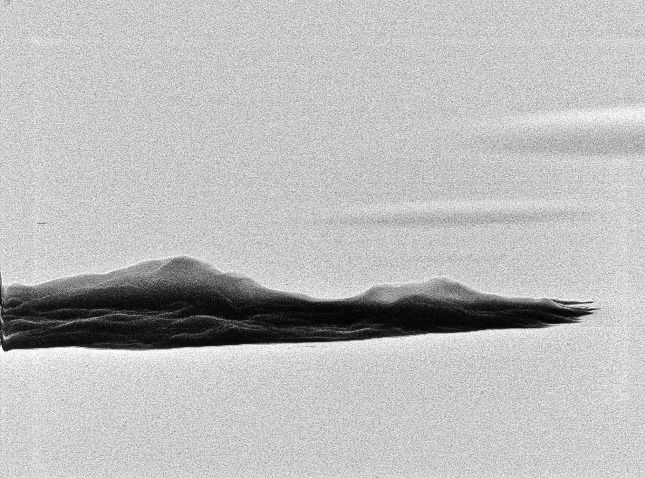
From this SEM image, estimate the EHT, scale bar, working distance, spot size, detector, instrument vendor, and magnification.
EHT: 30 kV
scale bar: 5000 nm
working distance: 22.4 mm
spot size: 3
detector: SE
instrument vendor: FEI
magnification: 6.177 K X